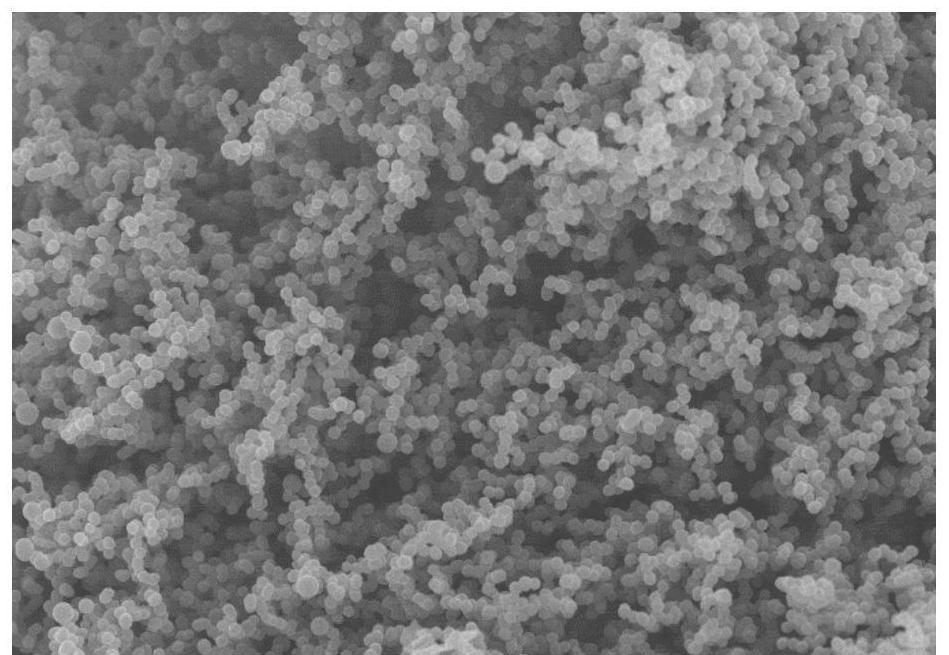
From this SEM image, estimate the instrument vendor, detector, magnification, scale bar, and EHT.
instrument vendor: Hitachi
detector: SE(M)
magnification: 10 K X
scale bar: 5000 nm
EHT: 5 kV